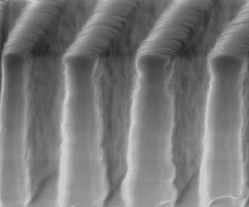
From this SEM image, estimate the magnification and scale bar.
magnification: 30 K X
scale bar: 400 nm